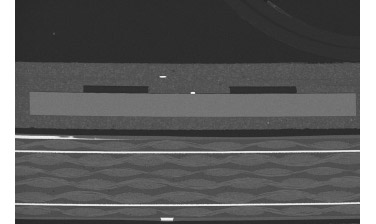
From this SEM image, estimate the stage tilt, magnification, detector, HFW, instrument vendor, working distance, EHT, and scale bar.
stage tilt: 0°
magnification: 0.08 K X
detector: T1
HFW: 5180 µm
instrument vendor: FEI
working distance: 51.4 mm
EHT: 30 kV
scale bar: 2000000 nm